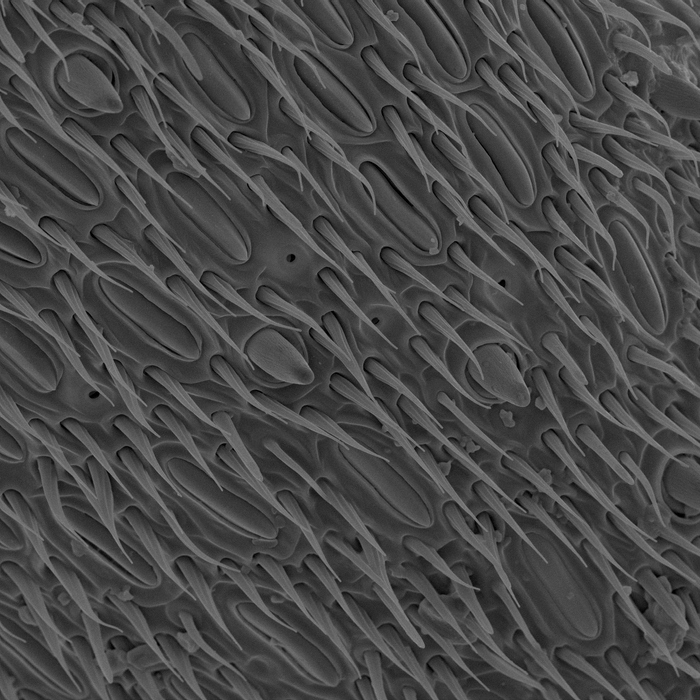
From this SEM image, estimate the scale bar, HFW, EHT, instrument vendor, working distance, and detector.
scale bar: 20000 nm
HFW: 100 µm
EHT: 10 kV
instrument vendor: Tescan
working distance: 6 mm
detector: SE